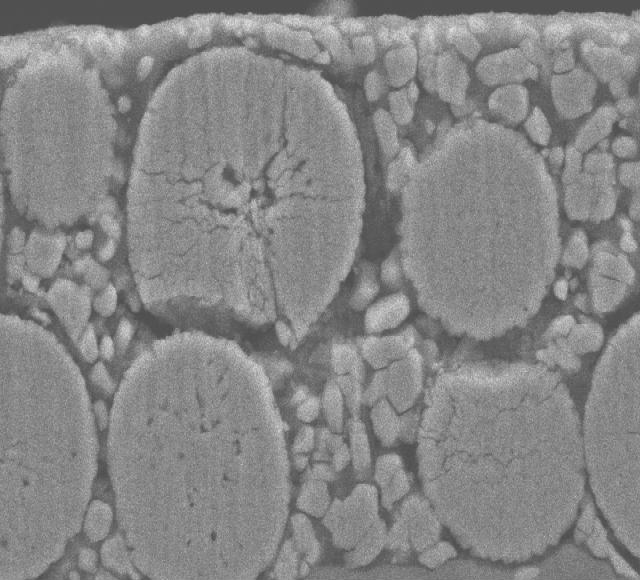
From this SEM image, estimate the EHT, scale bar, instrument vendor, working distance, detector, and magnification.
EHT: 15 kV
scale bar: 5000 nm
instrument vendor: JEOL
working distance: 8 mm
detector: SEI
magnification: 3 K X